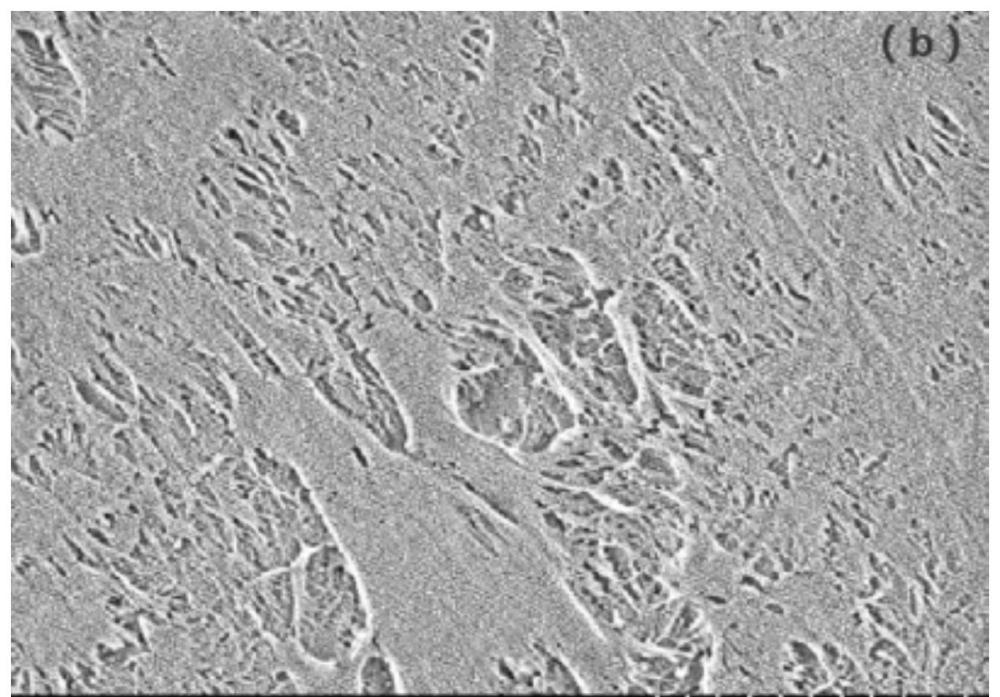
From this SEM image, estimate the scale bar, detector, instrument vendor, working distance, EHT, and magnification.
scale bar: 2000 nm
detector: SE+BSE(U)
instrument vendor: Hitachi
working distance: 3.6 mm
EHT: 1 kV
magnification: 20 K X